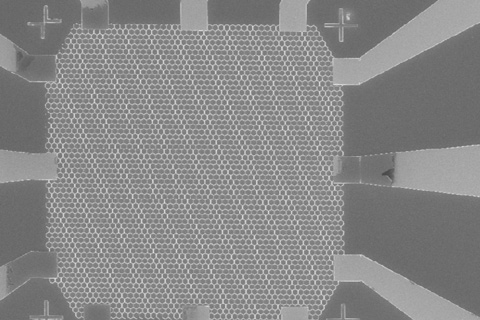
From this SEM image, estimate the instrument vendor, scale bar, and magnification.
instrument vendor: Zeiss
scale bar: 10000 nm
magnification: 0.75 K X